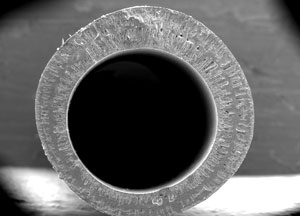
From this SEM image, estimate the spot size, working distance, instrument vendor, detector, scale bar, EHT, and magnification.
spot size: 4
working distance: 7.2 mm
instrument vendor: FEI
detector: GSE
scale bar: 500000 nm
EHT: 25 kV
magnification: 0.05 K X